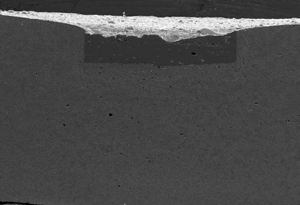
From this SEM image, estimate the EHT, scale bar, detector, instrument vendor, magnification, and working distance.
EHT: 15 kV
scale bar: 1000000 nm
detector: SE(U)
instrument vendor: Hitachi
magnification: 0.035 K X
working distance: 11.2 mm